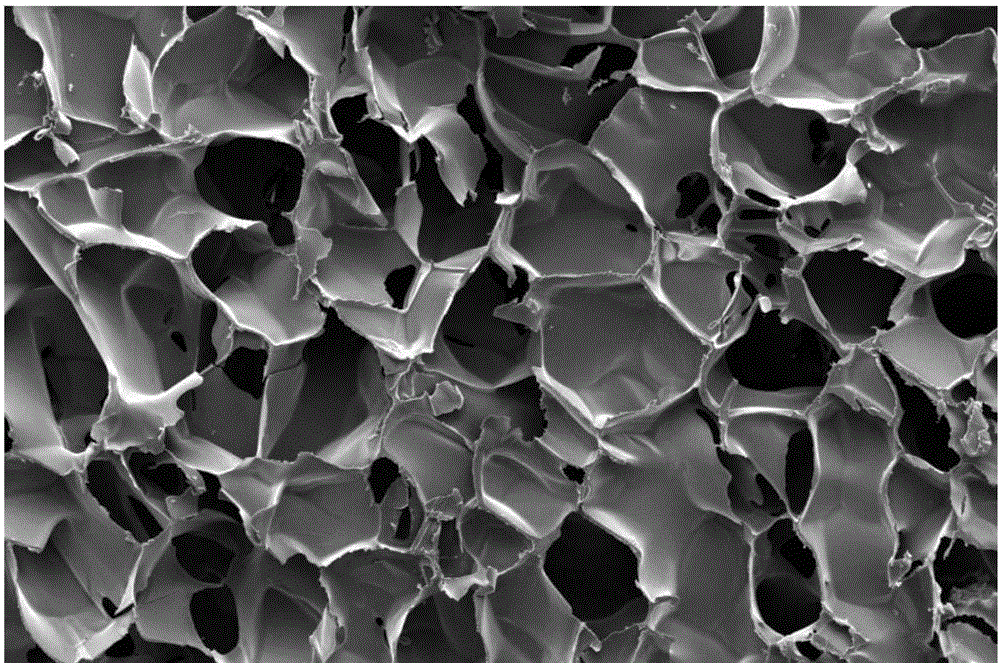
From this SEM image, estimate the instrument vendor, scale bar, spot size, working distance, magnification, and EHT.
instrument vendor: FEI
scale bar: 1000000 nm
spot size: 4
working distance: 14.3 mm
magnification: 0.2 K X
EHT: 10 kV